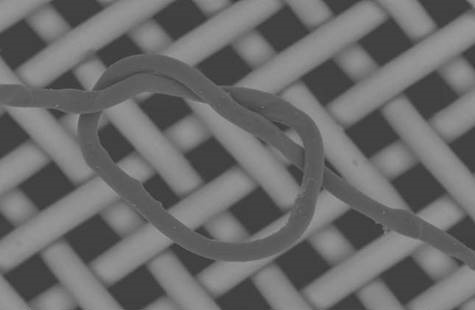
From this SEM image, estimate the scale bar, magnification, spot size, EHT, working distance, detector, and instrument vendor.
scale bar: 100000 nm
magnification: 0.3 K X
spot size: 5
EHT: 20 kV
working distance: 11.6 mm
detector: BSE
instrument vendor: FEI